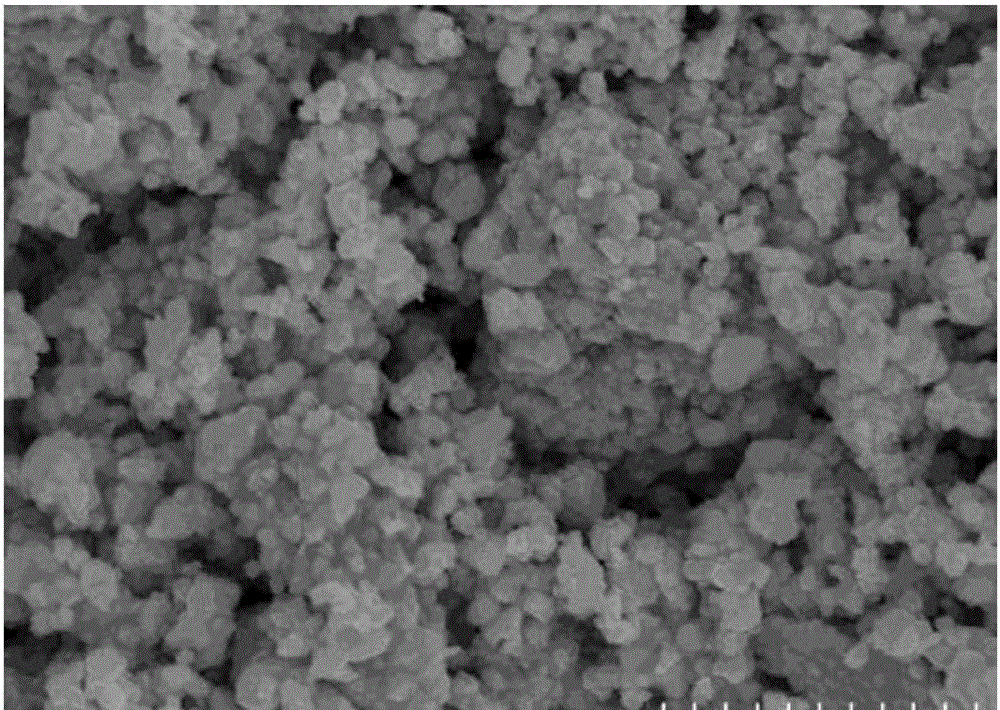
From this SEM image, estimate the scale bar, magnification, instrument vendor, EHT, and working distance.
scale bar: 2000 nm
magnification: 20 K X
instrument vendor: Hitachi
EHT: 15 kV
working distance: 9 mm